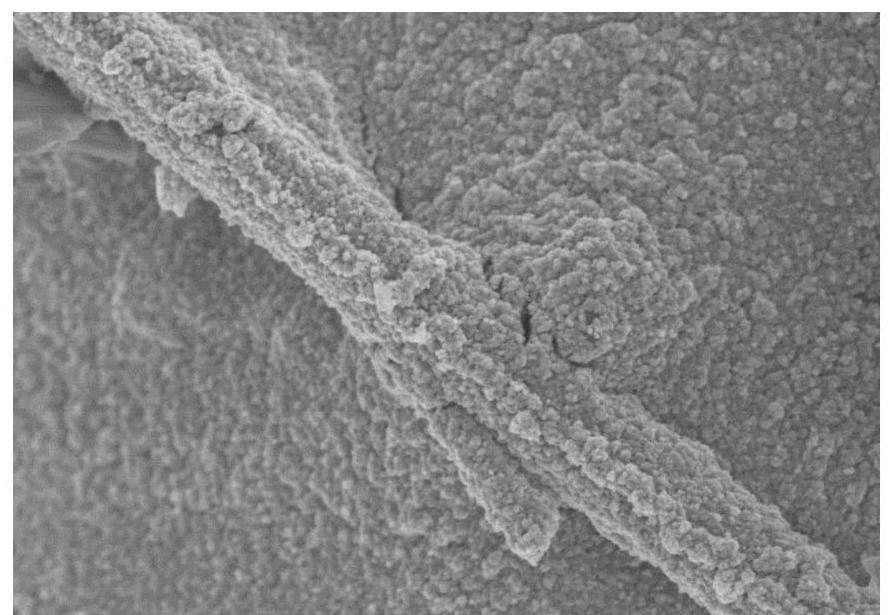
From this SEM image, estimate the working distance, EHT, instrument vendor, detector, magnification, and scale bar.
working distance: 8.2 mm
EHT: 5 kV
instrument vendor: Hitachi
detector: SE(M)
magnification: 10 K X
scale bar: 5000 nm